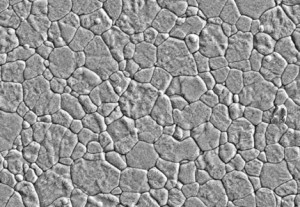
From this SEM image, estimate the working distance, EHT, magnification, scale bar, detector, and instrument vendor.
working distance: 16 mm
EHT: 5 kV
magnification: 10 K X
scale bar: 5000 nm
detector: SE(L)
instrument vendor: Hitachi